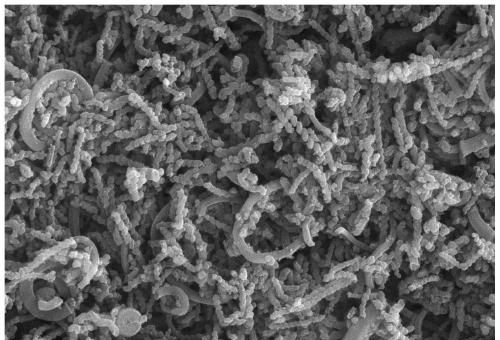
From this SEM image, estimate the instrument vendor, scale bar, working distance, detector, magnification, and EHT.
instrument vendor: Zeiss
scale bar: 200 nm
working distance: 4.1 mm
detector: InlensDuo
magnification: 50 K X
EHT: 10 kV